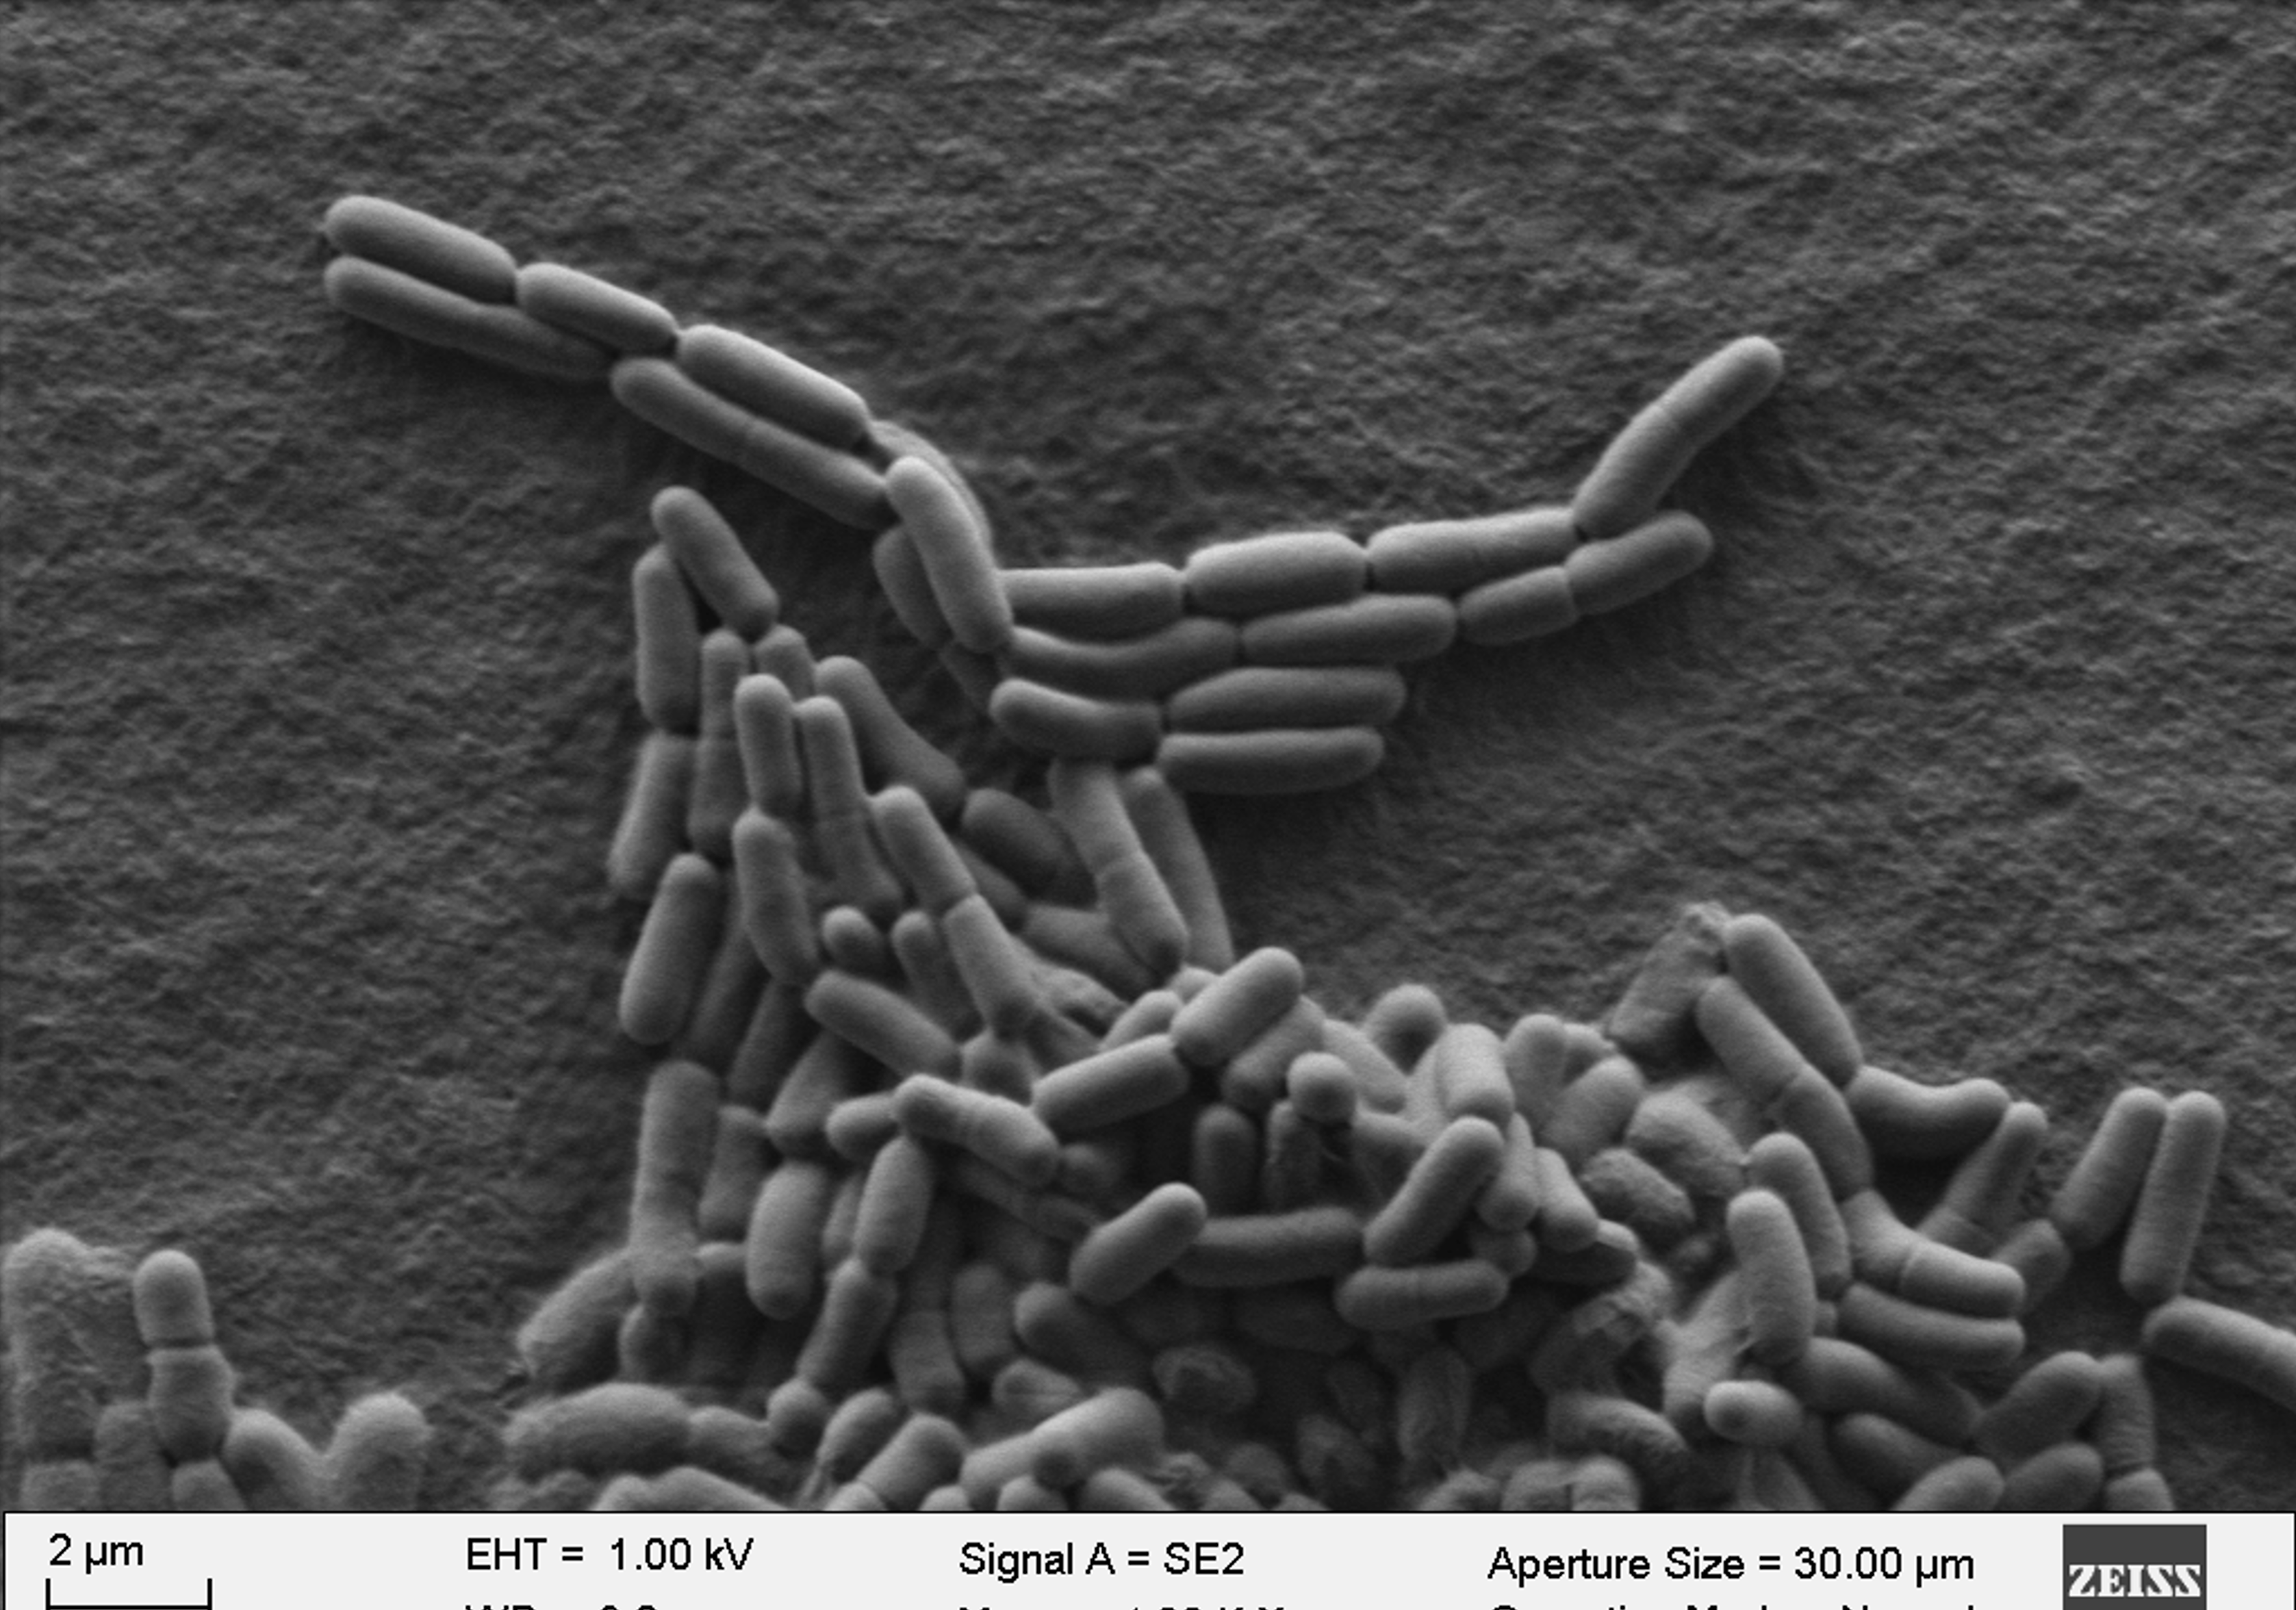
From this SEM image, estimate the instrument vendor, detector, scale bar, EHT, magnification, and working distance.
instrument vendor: Zeiss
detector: SE2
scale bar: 2000 nm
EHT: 1 kV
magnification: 4 K X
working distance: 3.2 mm